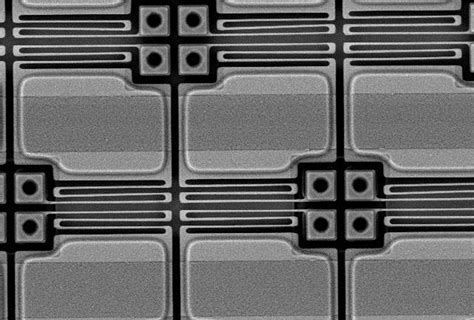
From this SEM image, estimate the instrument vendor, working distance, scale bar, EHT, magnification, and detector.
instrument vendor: Hitachi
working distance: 9 mm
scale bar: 20000 nm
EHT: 7 kV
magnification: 2.5 K X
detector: YAGBSE